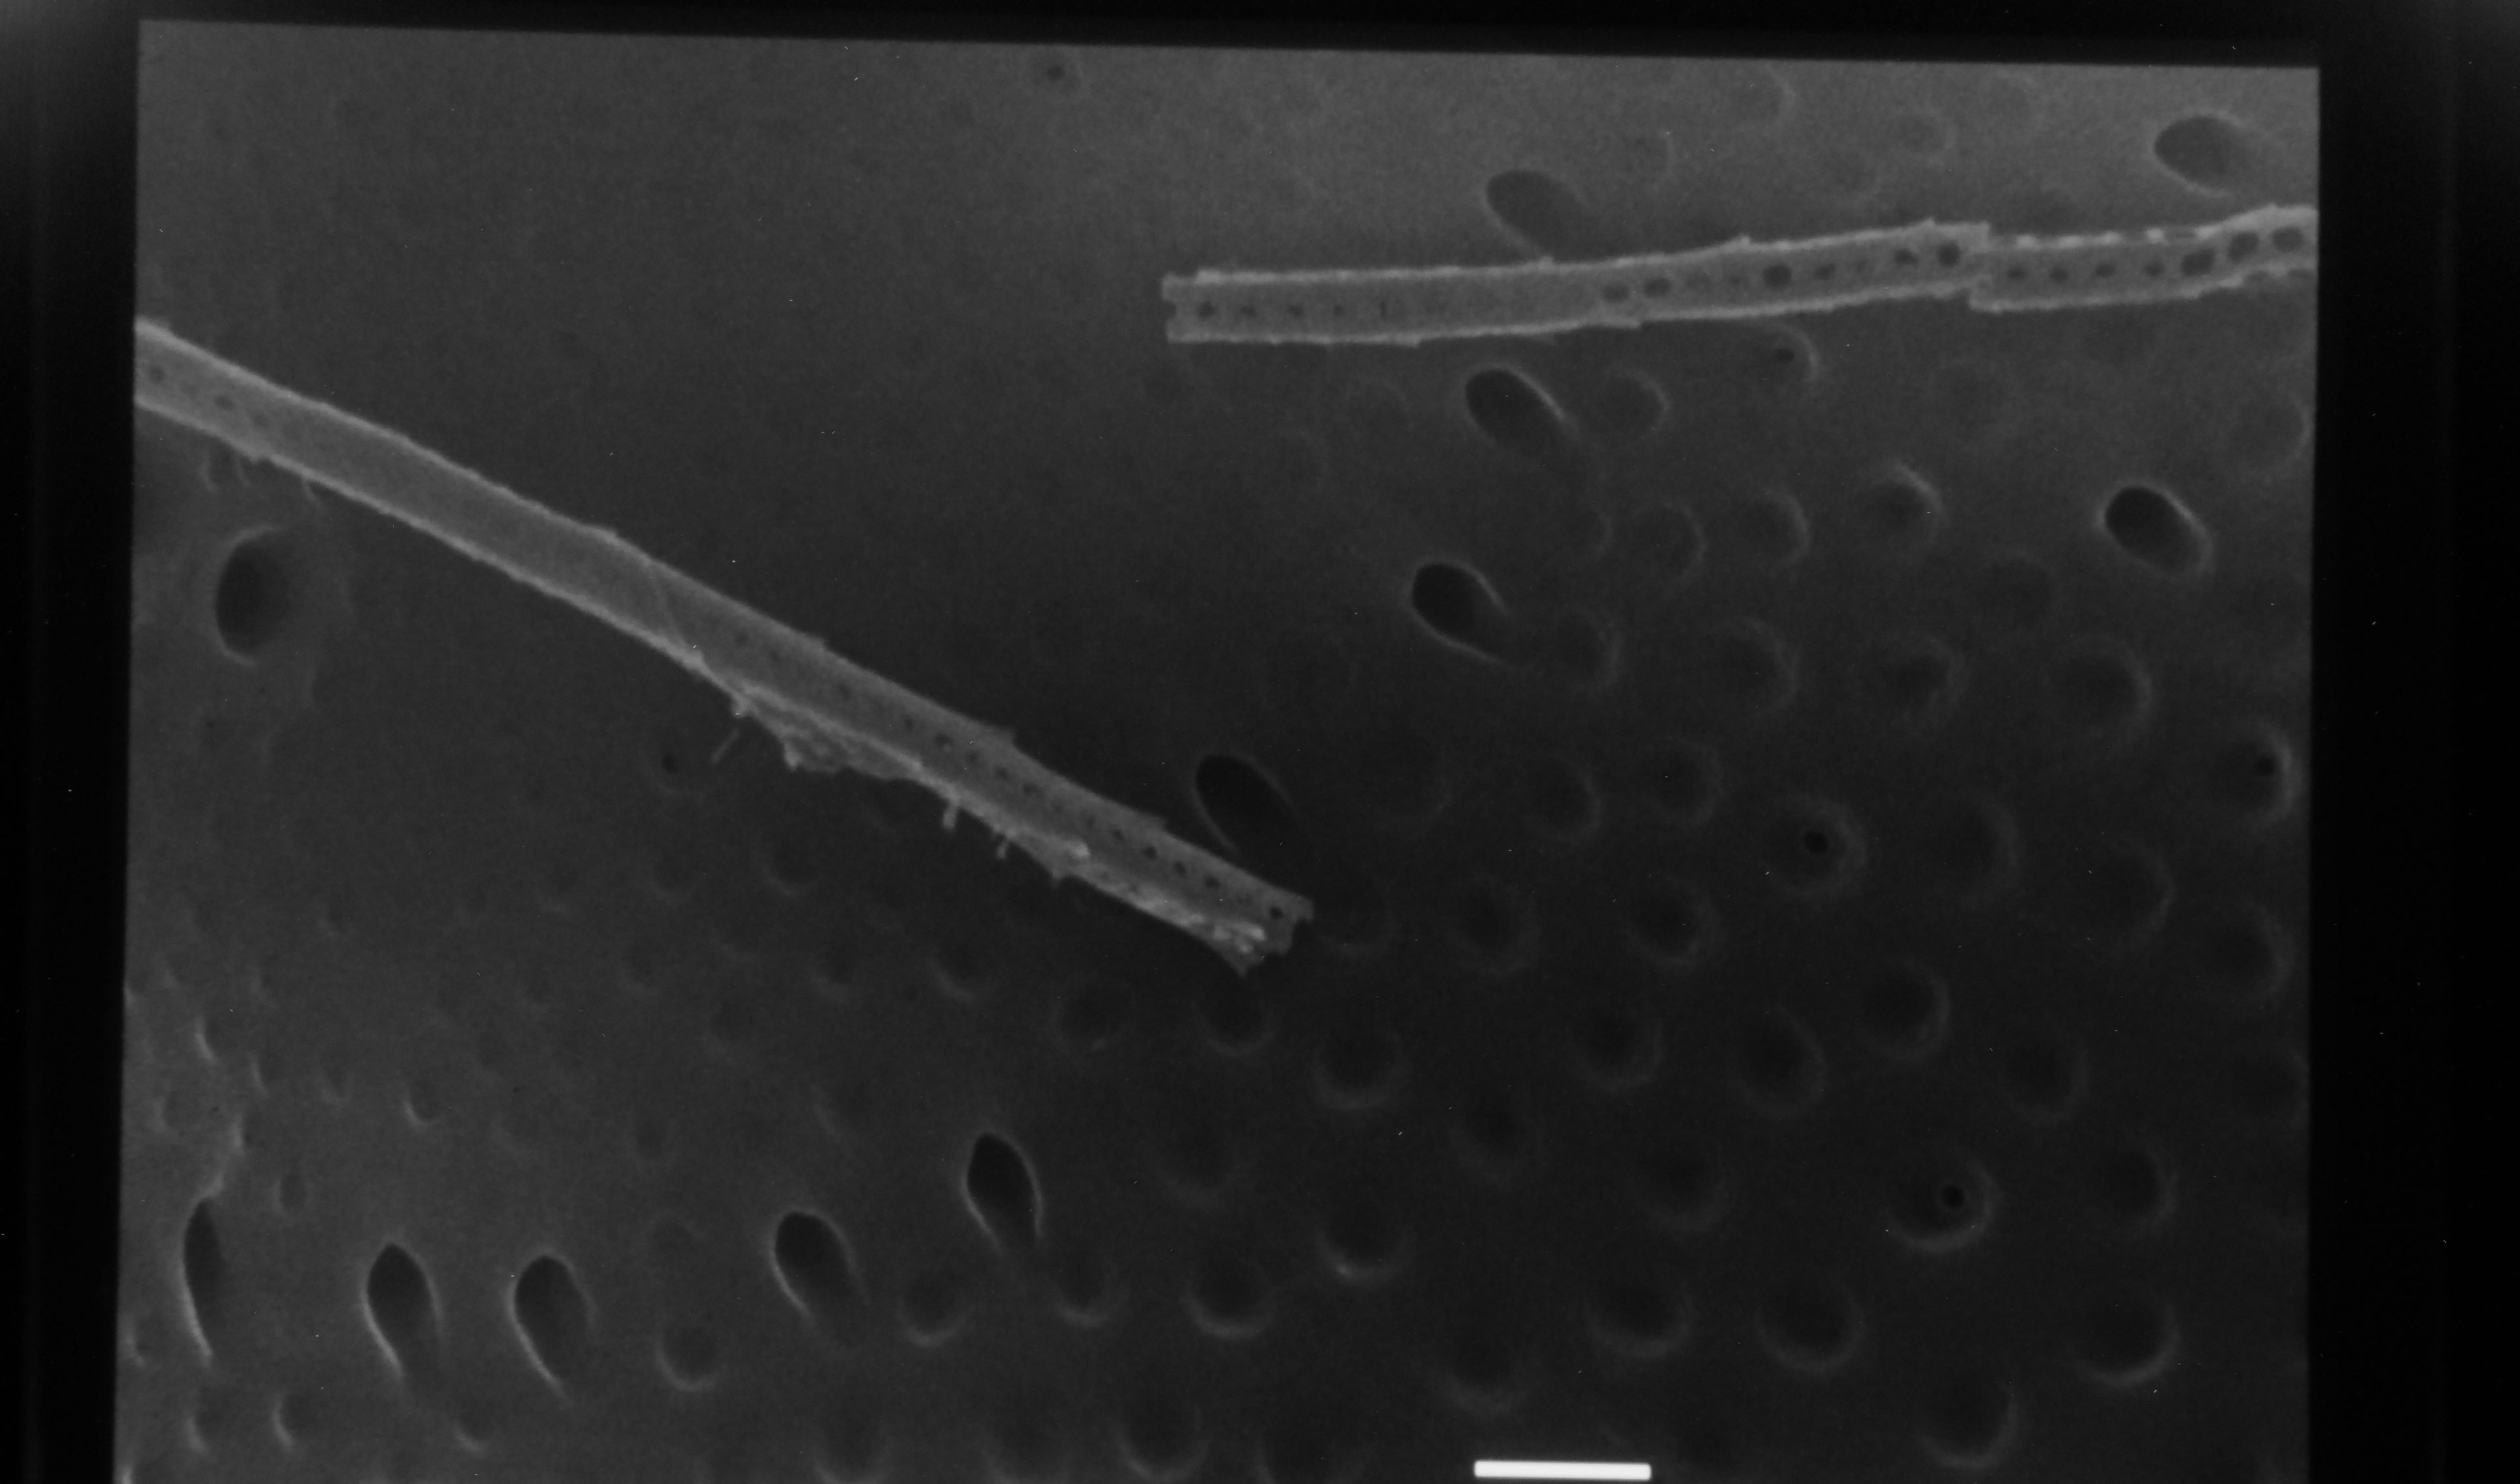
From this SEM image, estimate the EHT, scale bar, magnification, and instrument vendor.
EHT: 25 kV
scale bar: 1000 nm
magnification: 10 K X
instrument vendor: JEOL